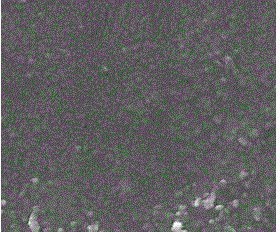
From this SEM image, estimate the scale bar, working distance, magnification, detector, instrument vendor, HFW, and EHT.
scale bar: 4000 nm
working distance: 5.1 mm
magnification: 20 K X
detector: ETD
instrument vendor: FEI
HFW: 14.9 µm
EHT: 20 kV